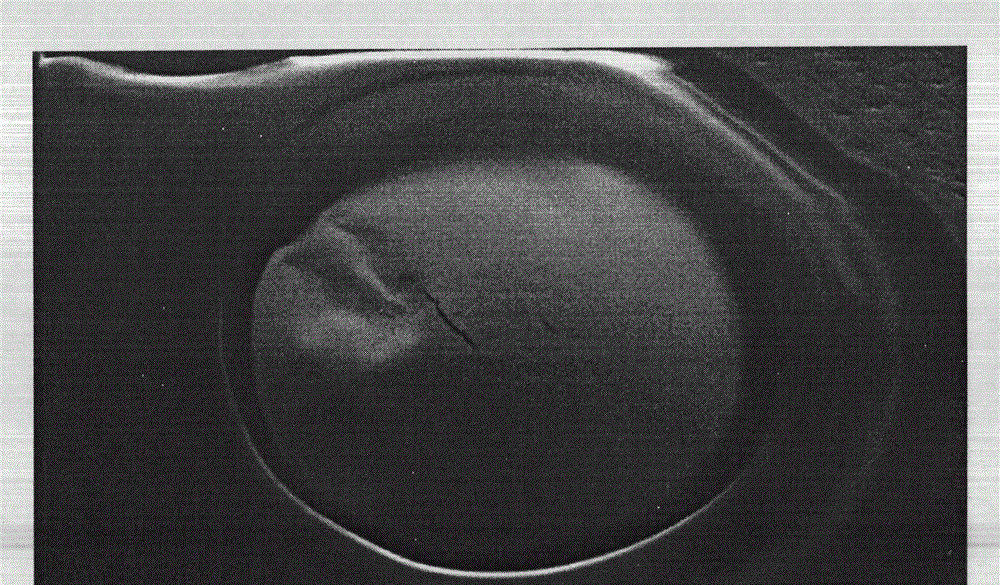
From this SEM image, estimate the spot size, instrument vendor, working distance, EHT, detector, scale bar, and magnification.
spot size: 4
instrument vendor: FEI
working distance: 11 mm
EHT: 20 kV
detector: SE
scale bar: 100000 nm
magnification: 0.2 K X